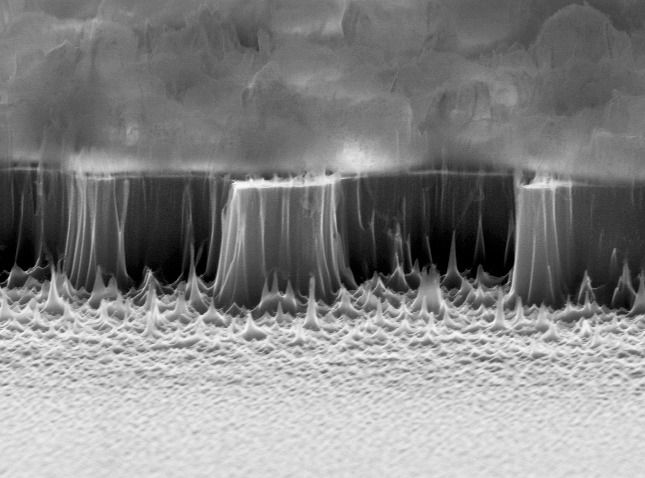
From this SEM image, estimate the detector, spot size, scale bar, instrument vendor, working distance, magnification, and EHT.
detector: SE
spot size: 4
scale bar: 2000 nm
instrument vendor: FEI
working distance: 8.6 mm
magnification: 12.836 K X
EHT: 30 kV